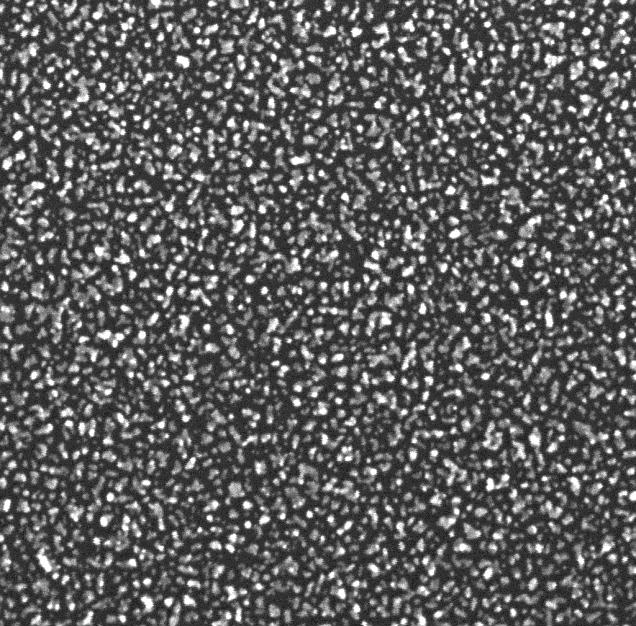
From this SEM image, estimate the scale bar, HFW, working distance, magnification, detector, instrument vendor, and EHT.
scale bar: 200 nm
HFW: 1 µm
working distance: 1.53 mm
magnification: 277 K X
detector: InBeam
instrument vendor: Tescan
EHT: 15 kV